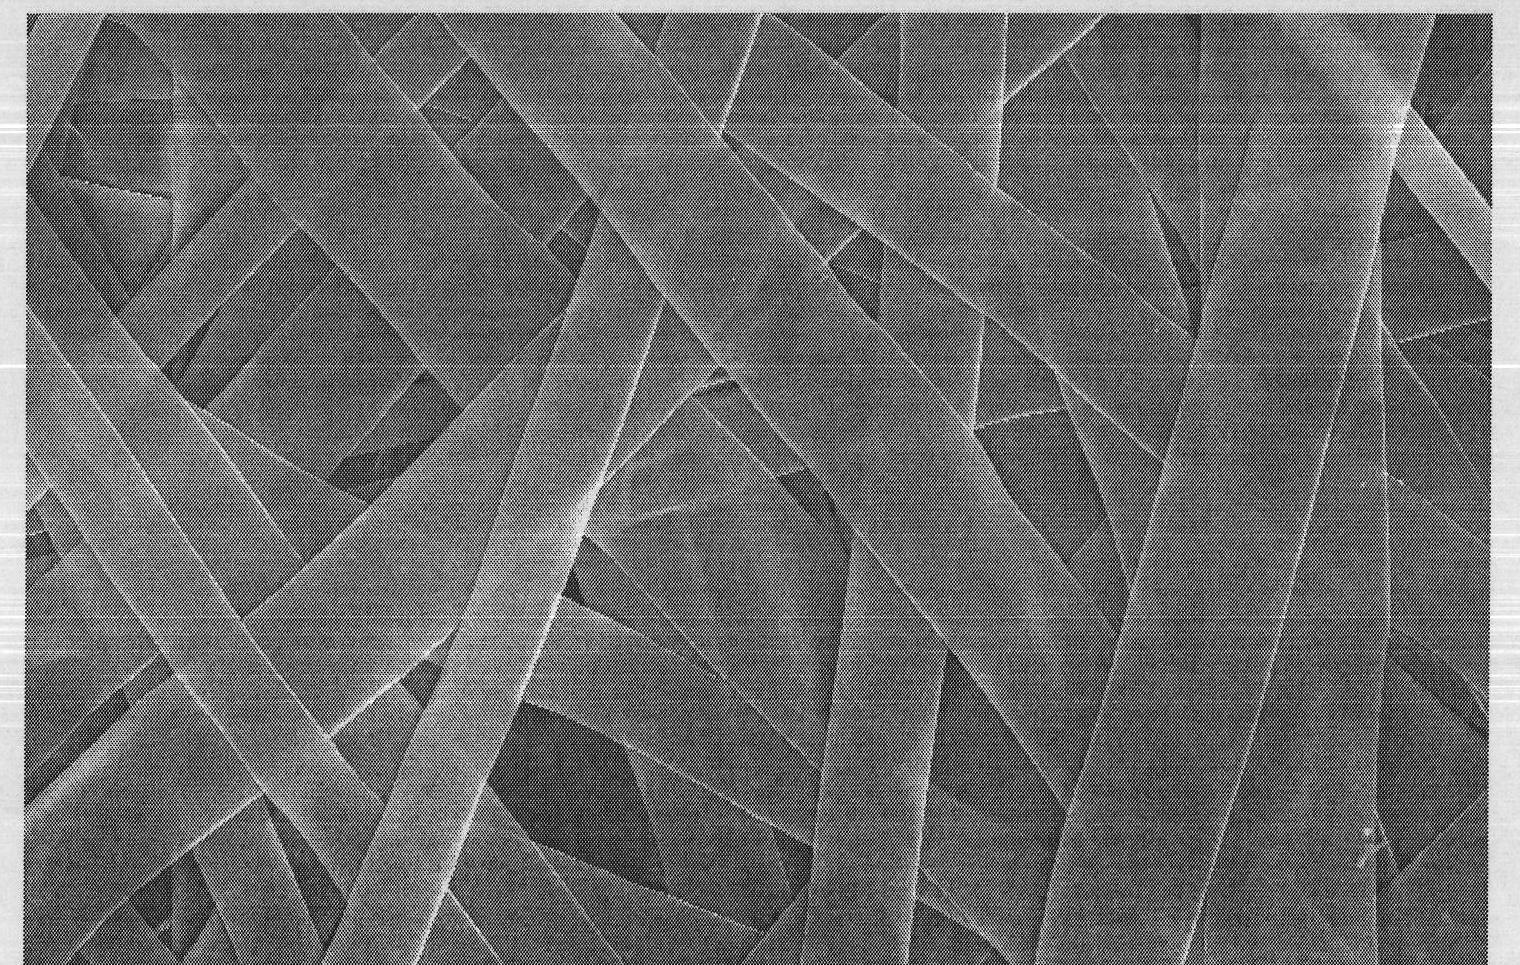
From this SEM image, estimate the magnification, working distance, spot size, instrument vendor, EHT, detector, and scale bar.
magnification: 1 K X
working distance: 10.8 mm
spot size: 4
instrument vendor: FEI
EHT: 20 kV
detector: SE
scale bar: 20000 nm